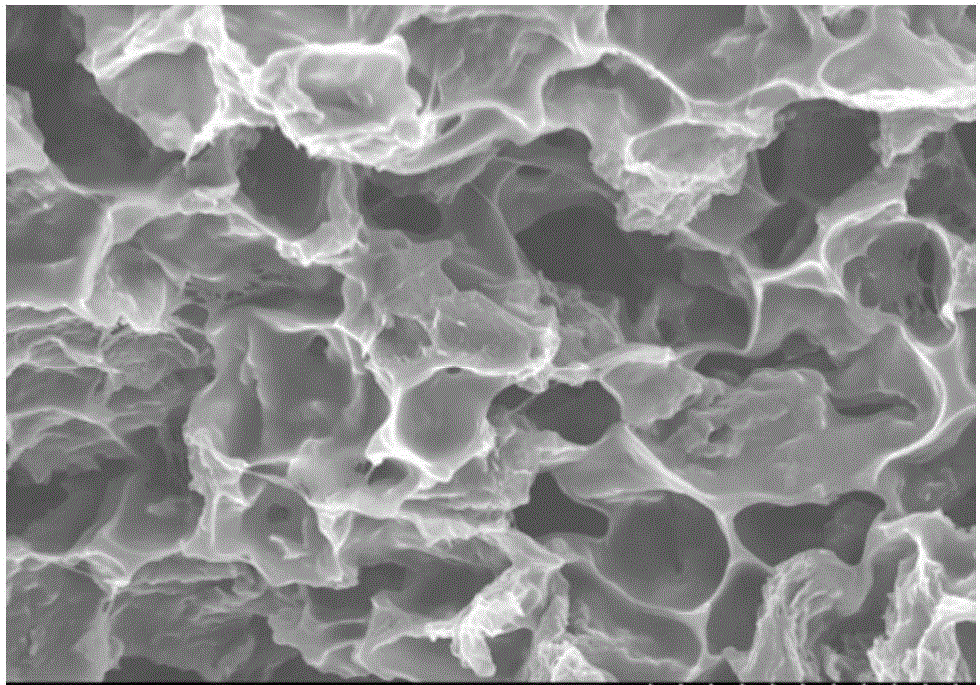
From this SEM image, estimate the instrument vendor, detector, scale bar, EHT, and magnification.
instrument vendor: Hitachi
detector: SE(U)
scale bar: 10000 nm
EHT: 8 kV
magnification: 4 K X